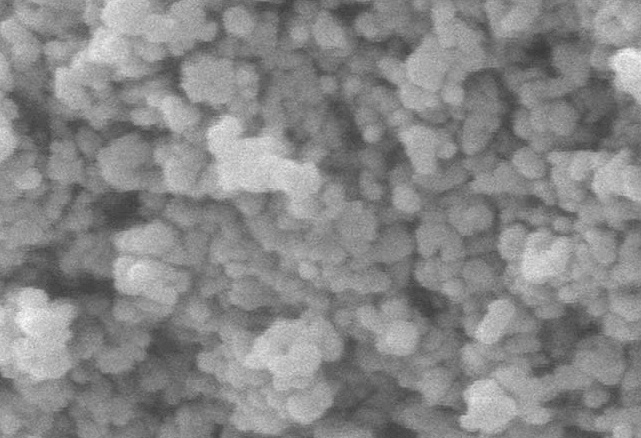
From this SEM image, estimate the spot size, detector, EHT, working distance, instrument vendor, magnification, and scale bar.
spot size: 1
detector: SE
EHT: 17 kV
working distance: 10.6 mm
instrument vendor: FEI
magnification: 30 K X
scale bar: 500 nm